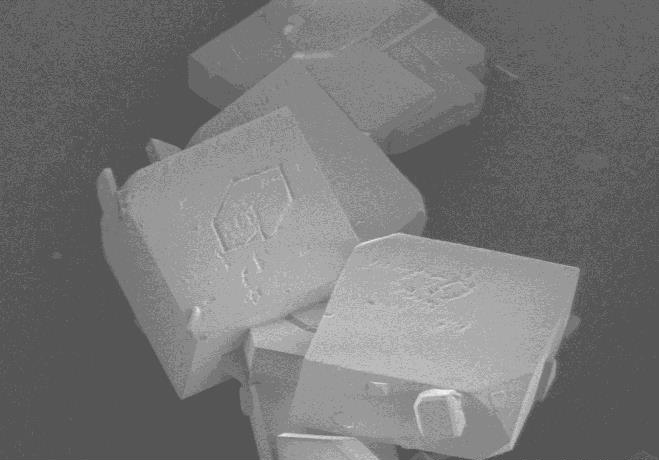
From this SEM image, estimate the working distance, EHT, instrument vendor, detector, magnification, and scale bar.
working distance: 6.9 mm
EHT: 1 kV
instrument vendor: Hitachi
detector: SE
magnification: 0.8 K X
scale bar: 50000 nm